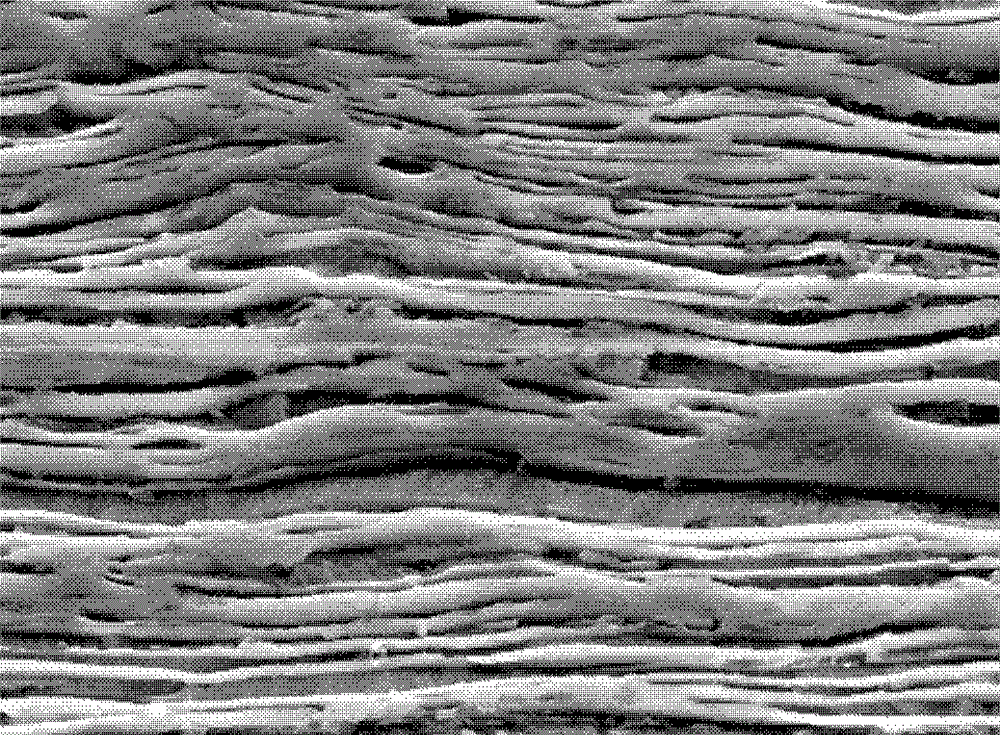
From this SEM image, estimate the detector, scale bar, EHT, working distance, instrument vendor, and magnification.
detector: SEI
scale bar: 1000 nm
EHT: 15 kV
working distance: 10.1 mm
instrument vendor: JEOL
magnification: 10 K X